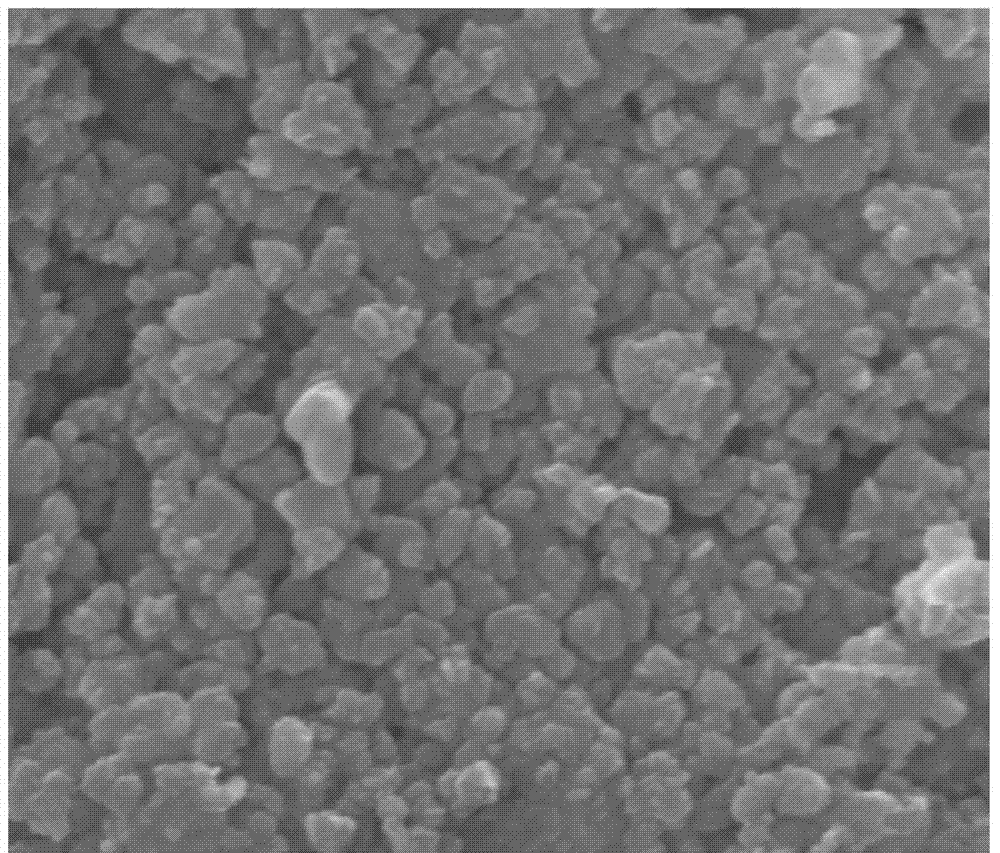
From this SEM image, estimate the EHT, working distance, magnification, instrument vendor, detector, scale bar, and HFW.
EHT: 20 kV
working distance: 12.8 mm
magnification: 100 K X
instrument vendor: FEI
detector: ETD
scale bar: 1000 nm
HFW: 2.98 µm